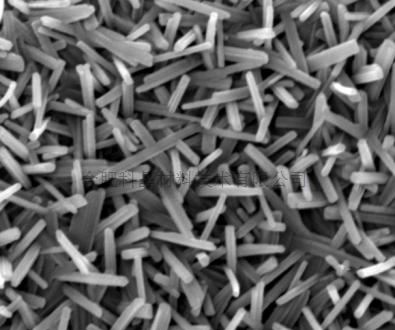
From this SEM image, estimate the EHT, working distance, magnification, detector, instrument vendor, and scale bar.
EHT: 20 kV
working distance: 6 mm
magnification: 40 K X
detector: TLD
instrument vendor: FEI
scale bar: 1000 nm